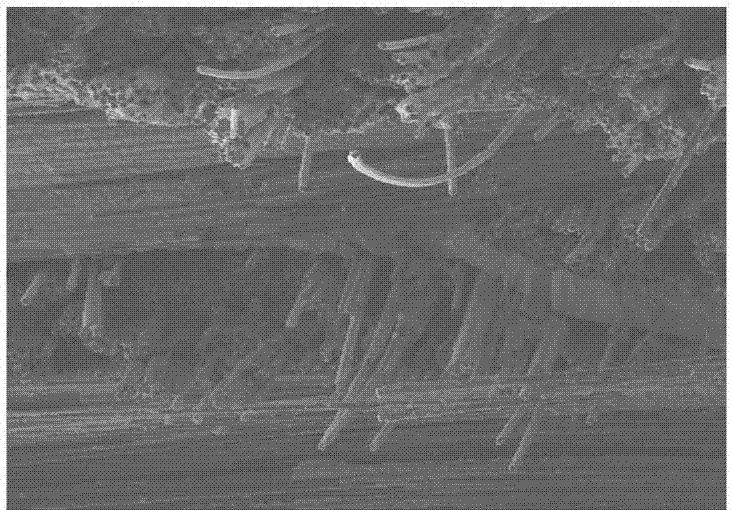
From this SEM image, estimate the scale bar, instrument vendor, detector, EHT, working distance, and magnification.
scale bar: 200000 nm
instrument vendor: Hitachi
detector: SE(M)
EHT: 10 kV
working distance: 11.6 mm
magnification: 0.2 K X